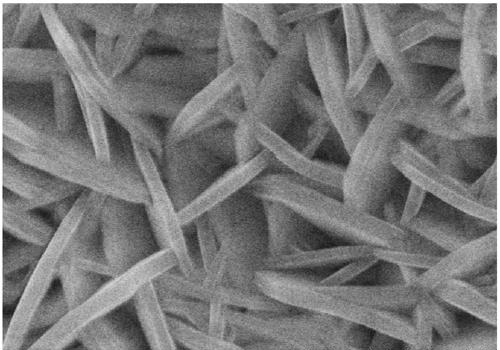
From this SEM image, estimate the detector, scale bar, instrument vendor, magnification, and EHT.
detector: SE(U)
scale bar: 1000 nm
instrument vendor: Hitachi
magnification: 50 K X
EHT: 15 kV